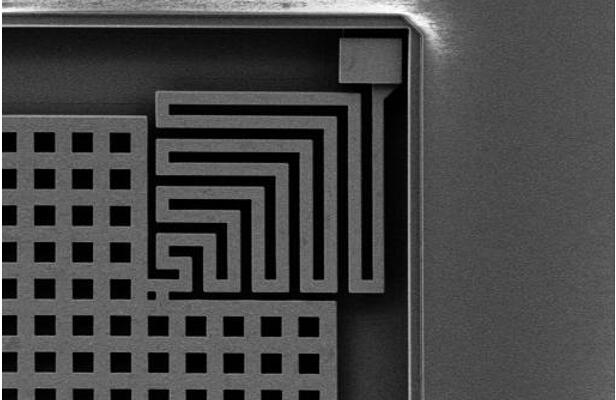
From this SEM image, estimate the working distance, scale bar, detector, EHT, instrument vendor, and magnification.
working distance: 5.7 mm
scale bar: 10000 nm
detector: SE2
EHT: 3 kV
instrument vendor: Zeiss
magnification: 4 K X